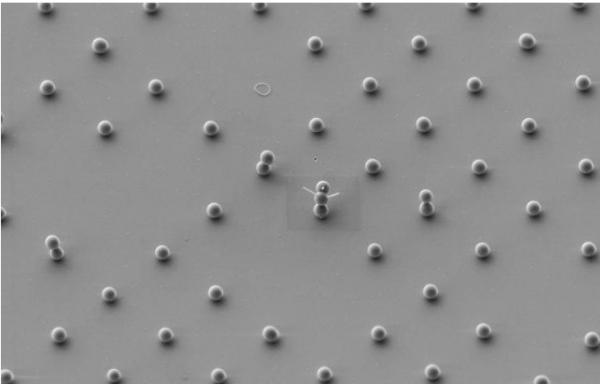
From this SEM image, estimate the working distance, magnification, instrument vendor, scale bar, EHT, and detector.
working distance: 4.4 mm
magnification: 3 K X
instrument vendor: Zeiss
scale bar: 2000 nm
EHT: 3 kV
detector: SE2